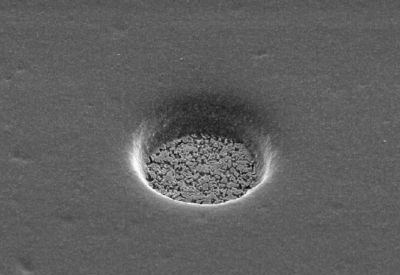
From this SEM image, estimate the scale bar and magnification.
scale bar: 10000 nm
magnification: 1 K X